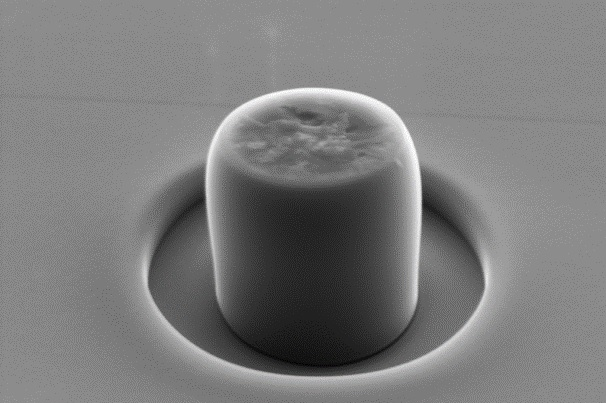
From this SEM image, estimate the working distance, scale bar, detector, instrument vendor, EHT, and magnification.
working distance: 6.2 mm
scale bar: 300 nm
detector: SE2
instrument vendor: Zeiss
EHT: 5 kV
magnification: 50 K X